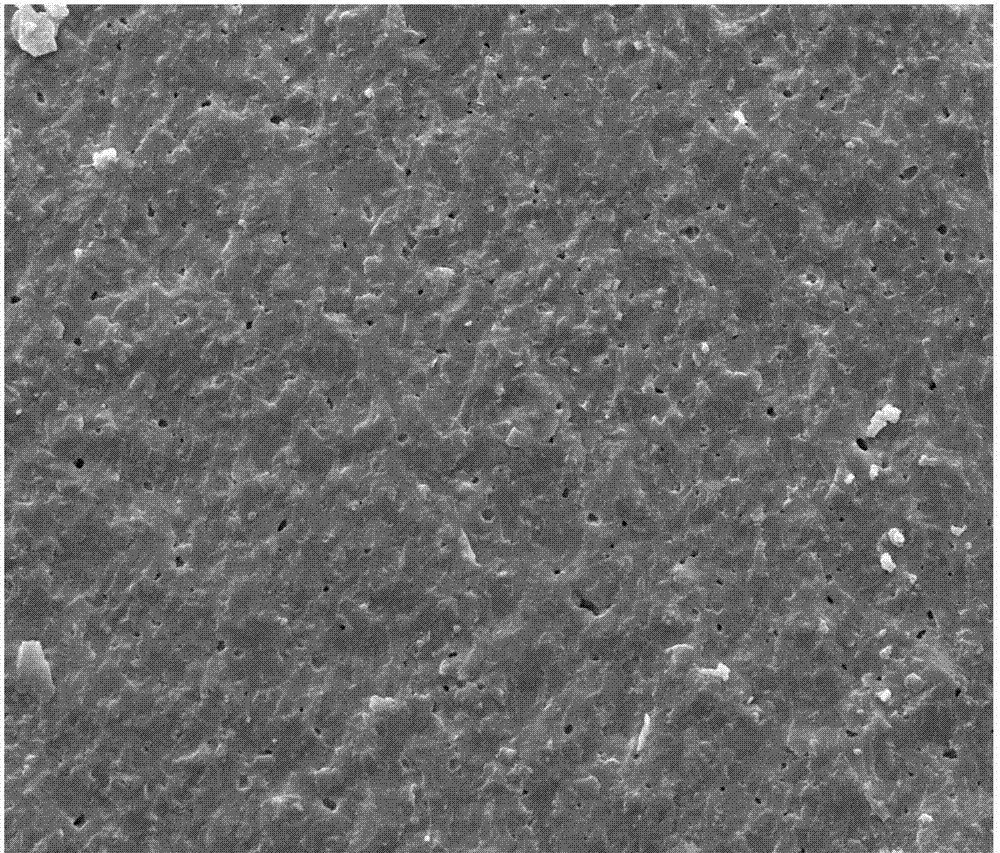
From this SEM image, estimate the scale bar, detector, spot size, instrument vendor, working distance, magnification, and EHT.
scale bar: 10000 nm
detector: ETD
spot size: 3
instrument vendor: FEI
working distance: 5.9 mm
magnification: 5 K X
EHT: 10 kV